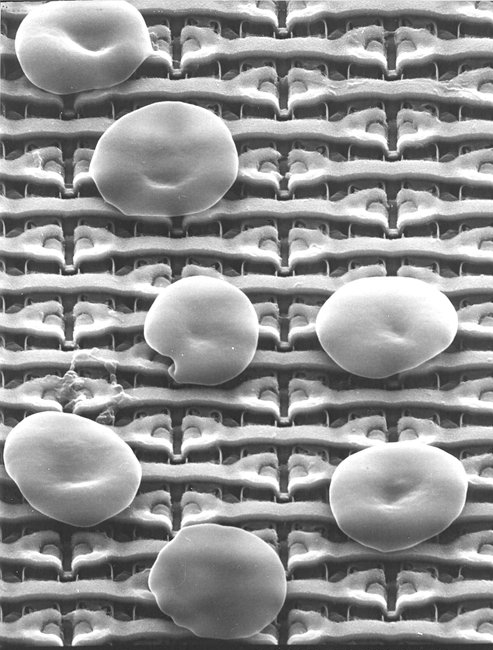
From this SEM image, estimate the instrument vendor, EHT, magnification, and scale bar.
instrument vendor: JEOL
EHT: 25 kV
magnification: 4 K X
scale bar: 7500 nm